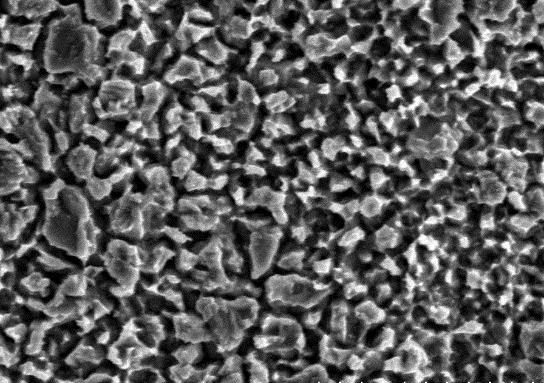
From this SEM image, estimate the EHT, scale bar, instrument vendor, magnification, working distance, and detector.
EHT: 15 kV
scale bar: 10000 nm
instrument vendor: Hitachi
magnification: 5 K X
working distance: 15.6 mm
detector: SE